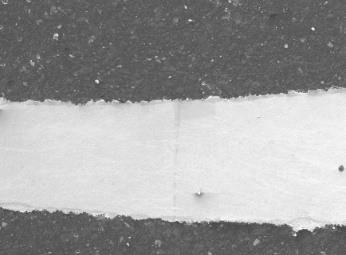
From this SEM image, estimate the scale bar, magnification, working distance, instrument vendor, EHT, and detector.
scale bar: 100000 nm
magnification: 0.05 K X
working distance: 13.7 mm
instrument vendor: JEOL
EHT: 15 kV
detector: LEI